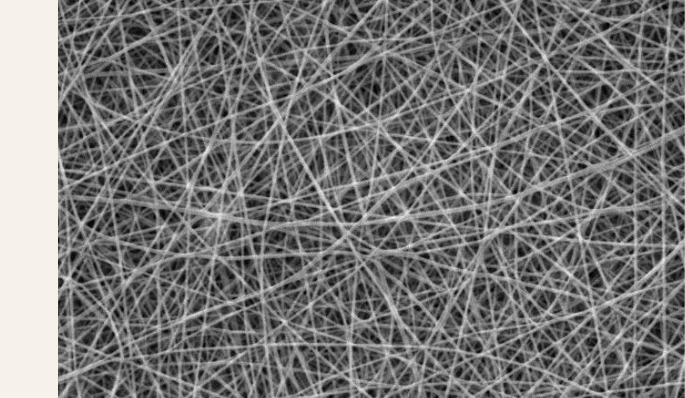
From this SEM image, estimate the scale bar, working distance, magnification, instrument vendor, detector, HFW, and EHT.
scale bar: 10000 nm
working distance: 16.29 mm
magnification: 5 K X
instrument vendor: Tescan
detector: SE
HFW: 55.4 µm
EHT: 20 kV